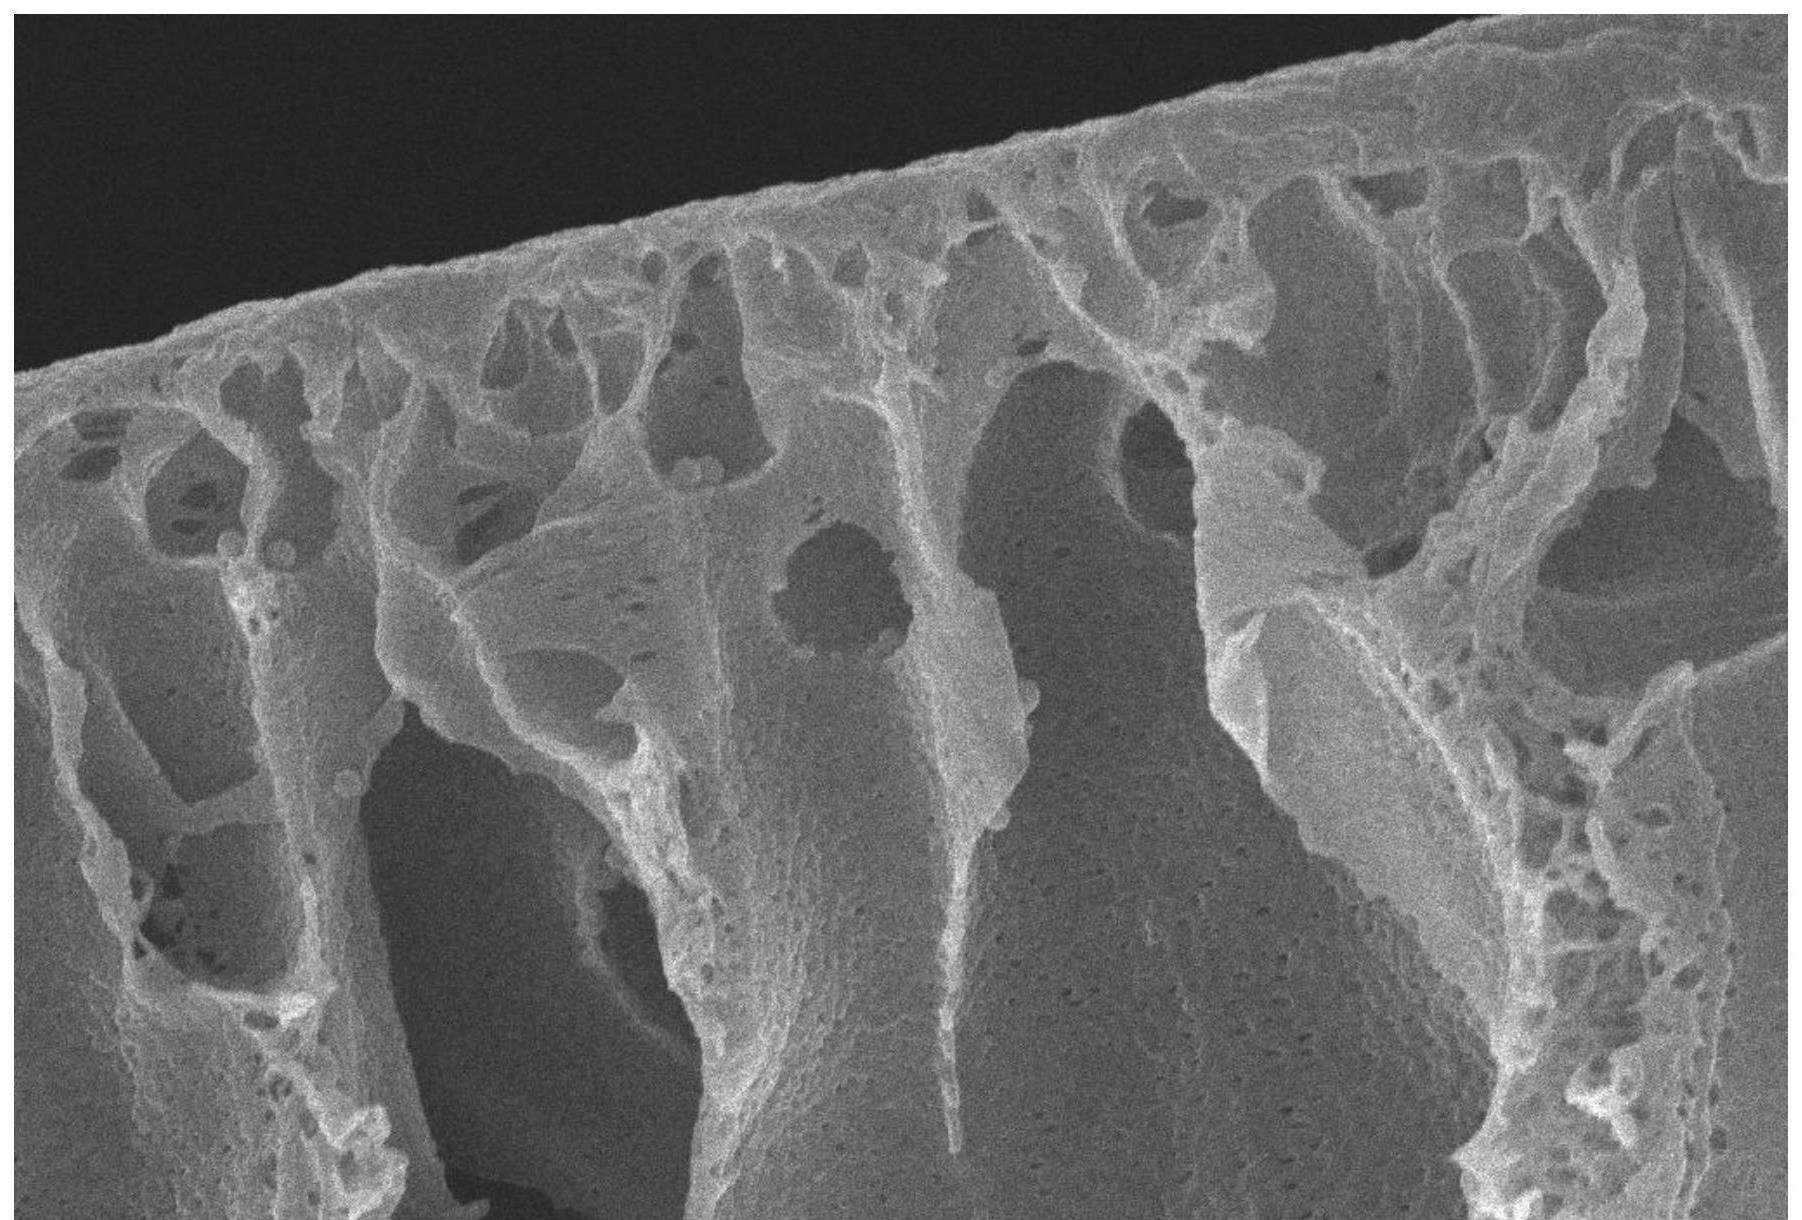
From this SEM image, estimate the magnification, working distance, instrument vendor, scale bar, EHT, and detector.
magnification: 10 K X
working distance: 8.6 mm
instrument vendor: Zeiss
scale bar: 1000 nm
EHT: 10 kV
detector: InLens